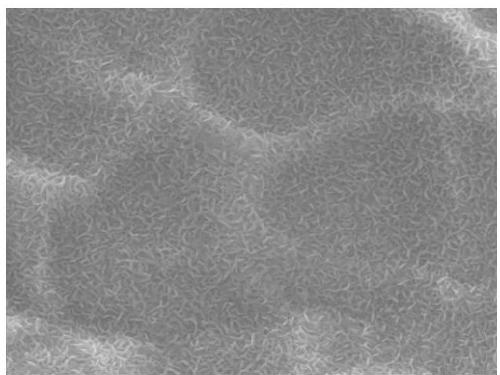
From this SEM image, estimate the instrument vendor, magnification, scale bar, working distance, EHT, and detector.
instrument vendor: JEOL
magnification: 20 K X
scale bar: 1000 nm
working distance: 11.3 mm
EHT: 10 kV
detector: LED